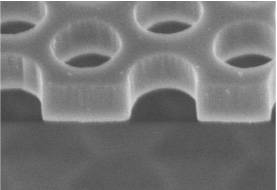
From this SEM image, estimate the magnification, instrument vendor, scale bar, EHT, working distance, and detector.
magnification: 100 K X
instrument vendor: Hitachi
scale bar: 500 nm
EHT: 2 kV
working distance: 3.7 mm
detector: SE(U)